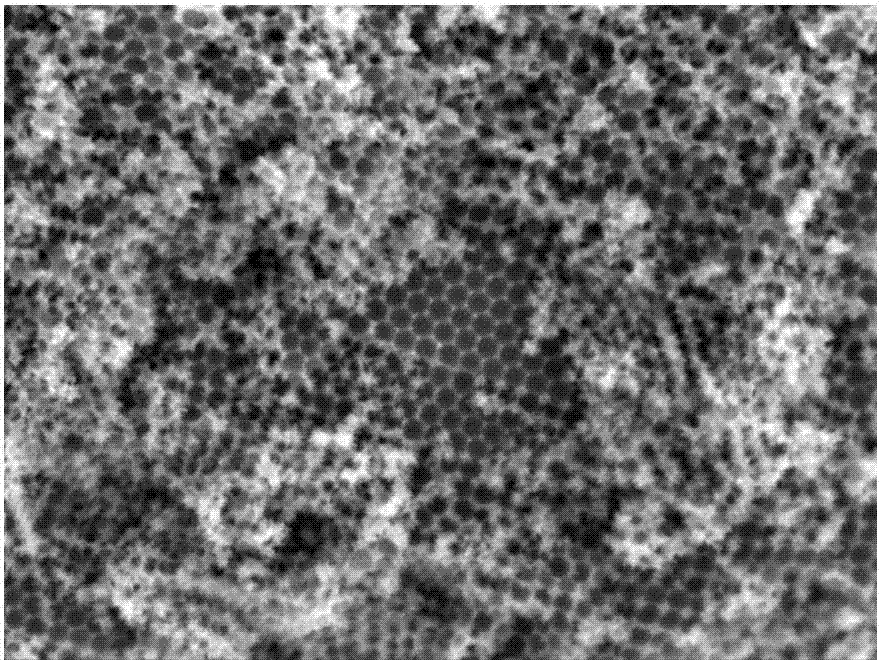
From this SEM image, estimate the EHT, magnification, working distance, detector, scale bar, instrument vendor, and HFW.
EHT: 5 kV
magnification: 60 K X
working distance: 6 mm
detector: InBeam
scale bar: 1000 nm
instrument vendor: Tescan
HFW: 4.21 µm